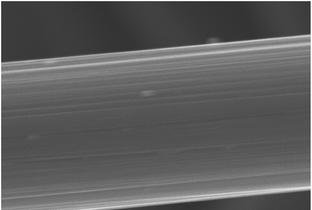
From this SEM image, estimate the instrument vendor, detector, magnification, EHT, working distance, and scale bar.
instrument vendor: Hitachi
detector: SE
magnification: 10 K X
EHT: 15 kV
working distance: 12.7 mm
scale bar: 5000 nm